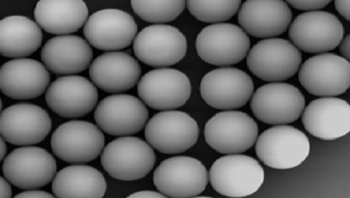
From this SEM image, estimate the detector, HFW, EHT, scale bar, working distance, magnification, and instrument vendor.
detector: SE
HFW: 13.8 µm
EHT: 10 kV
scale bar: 2000 nm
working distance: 8.63 mm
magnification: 20 K X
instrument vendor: Tescan